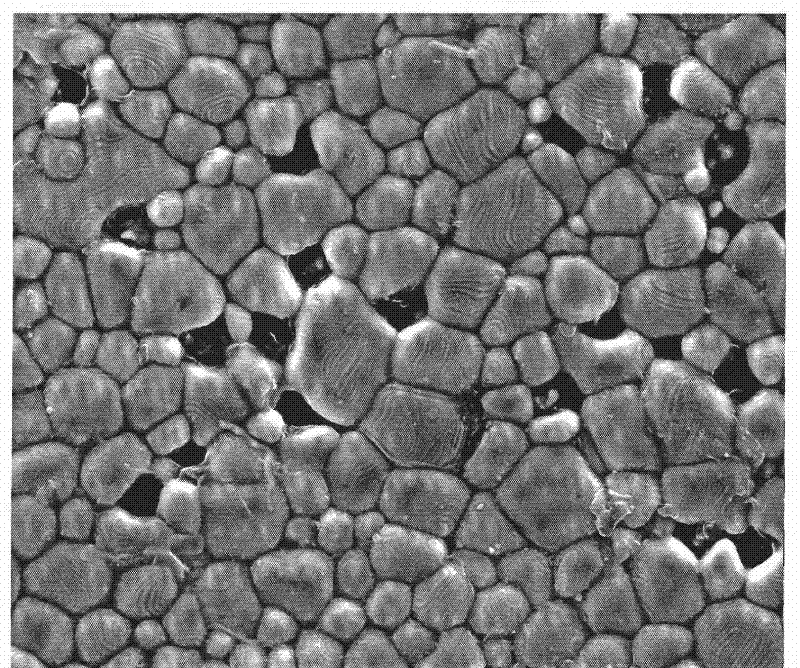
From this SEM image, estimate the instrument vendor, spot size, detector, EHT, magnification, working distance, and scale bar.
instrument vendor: FEI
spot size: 4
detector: ETD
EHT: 20 kV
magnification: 2.4 K X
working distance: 11.3 mm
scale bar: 20000 nm